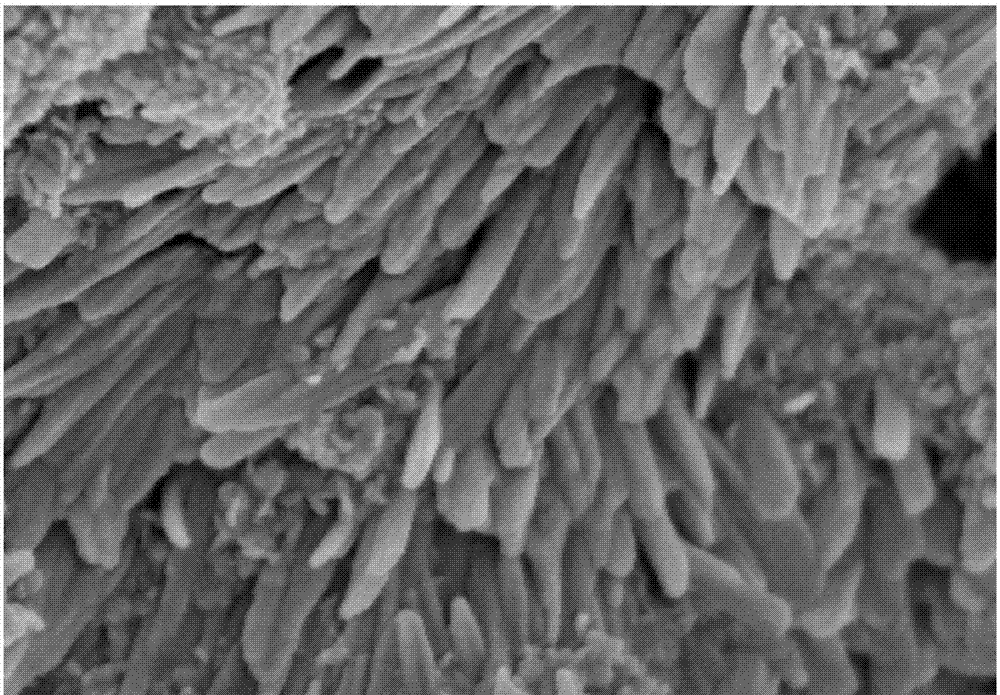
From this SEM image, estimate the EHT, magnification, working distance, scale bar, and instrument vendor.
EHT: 3 kV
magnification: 100 K X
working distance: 3 mm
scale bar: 500 nm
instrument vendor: Hitachi